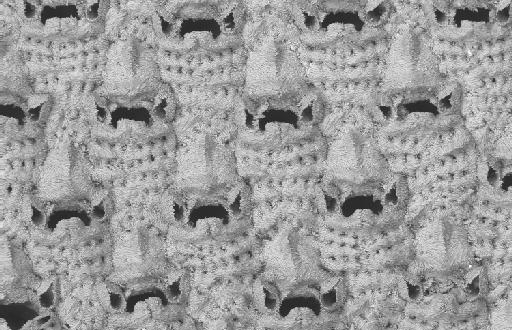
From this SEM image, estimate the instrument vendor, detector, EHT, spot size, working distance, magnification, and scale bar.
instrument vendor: Zeiss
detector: QBSD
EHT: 15 kV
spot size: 500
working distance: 15 mm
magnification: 0.25 K X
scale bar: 100000 nm